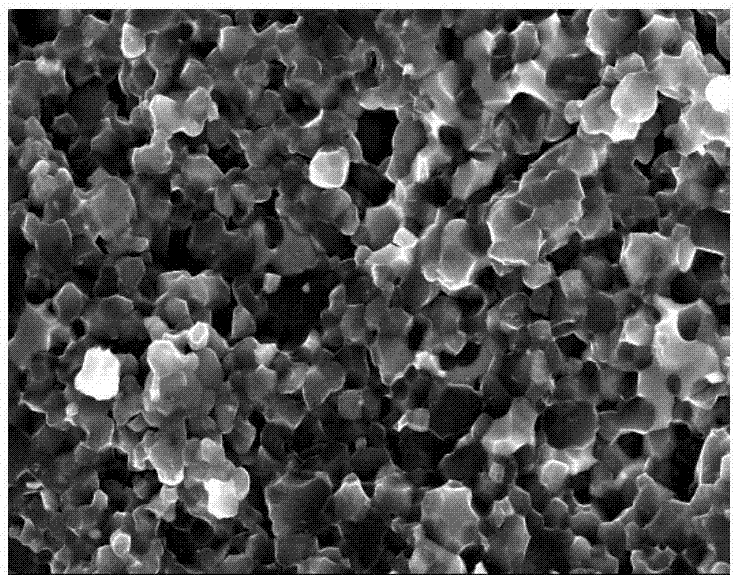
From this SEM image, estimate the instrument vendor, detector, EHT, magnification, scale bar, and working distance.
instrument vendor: Hitachi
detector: SE(M)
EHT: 15 kV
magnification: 10 K X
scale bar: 5000 nm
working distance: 9 mm